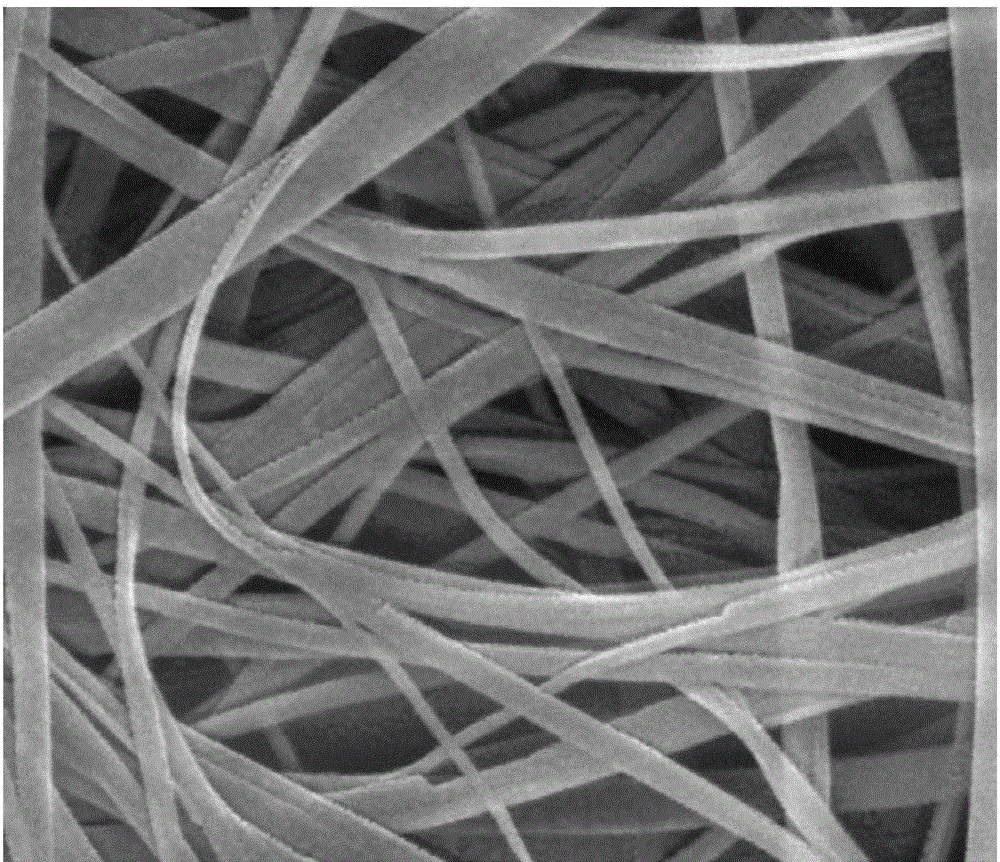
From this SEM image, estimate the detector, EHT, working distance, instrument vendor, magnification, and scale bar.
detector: SE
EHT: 20 kV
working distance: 6.1 mm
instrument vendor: FEI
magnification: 120 K X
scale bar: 500 nm